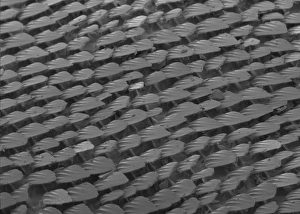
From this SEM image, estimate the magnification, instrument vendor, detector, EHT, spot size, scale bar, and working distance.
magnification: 0.037 K X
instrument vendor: FEI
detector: SE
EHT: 5 kV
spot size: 3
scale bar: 500000 nm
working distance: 9 mm